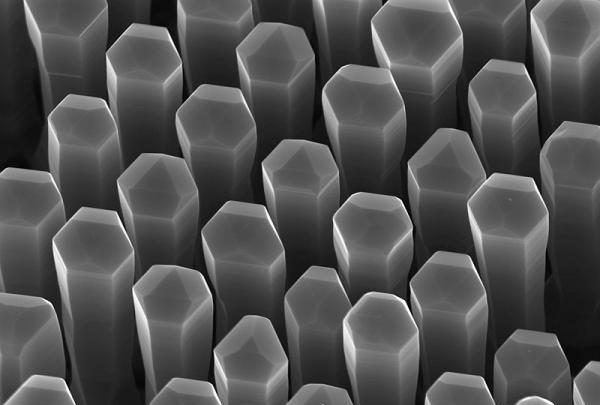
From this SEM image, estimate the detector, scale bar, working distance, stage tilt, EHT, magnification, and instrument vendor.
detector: InLens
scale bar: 200 nm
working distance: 3 mm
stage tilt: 30°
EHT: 10 kV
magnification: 50 K X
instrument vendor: Zeiss